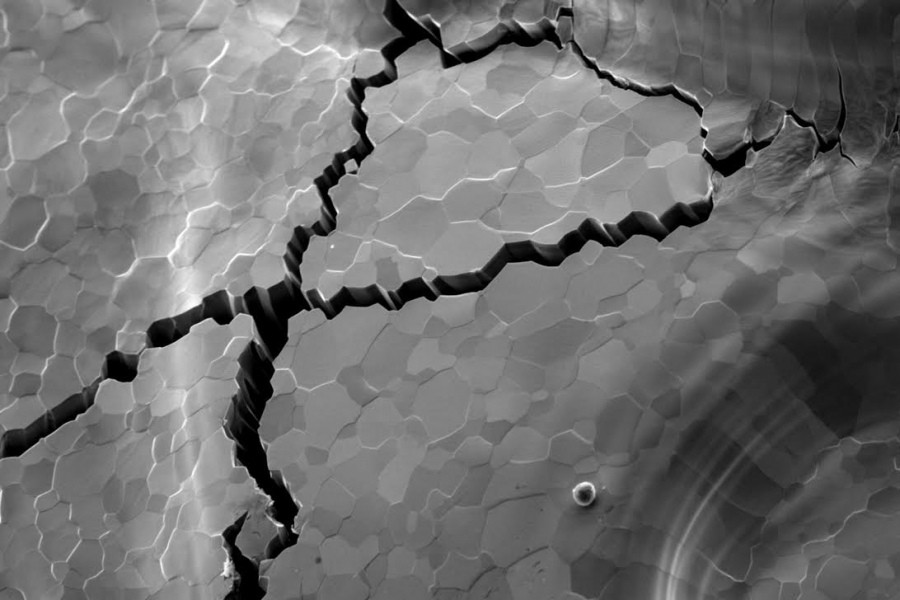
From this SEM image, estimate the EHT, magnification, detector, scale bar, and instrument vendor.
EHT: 15 kV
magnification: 0.6 K X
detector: SEI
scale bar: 20000 nm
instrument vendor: JEOL